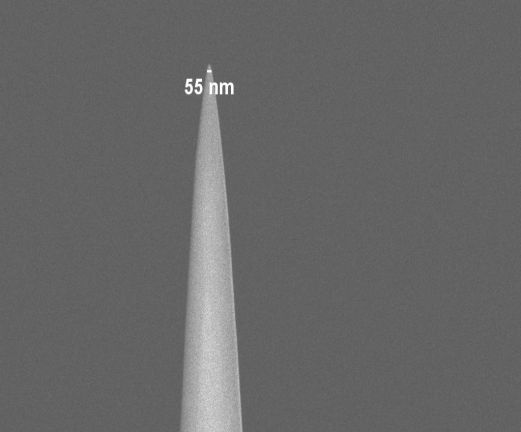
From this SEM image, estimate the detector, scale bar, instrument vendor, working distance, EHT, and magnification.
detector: InLens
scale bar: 2000 nm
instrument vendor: Zeiss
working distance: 4.9 mm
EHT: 2 kV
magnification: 20 K X